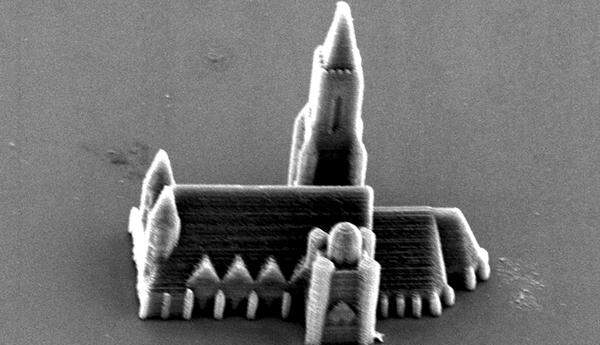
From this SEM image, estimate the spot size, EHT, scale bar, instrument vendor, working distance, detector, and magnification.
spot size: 4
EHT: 15 kV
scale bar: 20000 nm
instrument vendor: FEI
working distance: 27.5 mm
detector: SE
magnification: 0.859 K X